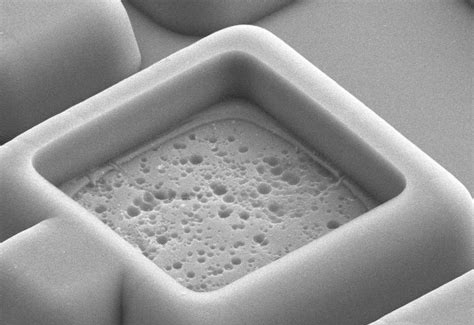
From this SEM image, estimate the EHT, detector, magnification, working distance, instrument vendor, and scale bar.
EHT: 10 kV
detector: SE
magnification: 7 K X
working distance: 34.8 mm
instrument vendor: Hitachi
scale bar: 5000 nm